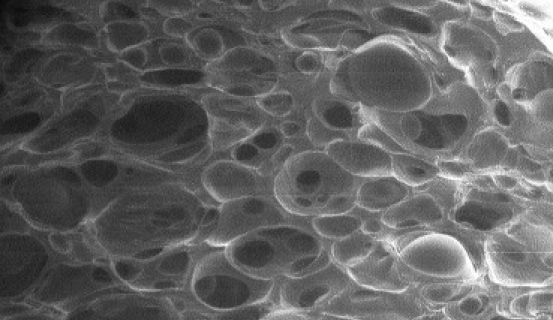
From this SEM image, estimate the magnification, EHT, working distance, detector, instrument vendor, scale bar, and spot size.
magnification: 0.025 K X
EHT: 30 kV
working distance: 12.1 mm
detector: GSE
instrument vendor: FEI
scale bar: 1000000 nm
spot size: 6